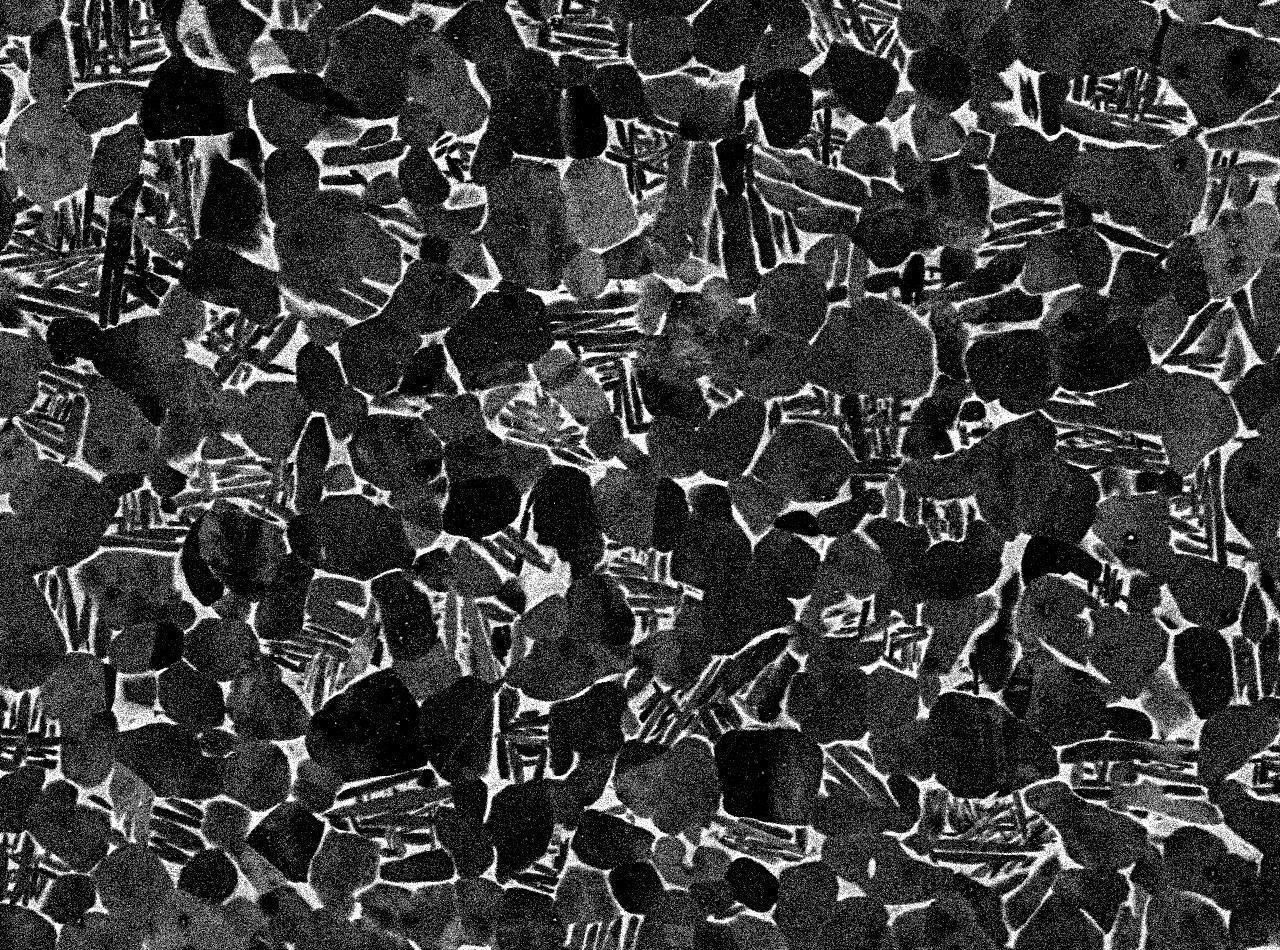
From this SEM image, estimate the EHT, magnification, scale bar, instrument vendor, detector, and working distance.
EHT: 15 kV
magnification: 1.1 K X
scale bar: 10000 nm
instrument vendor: JEOL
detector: COMPO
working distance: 10 mm